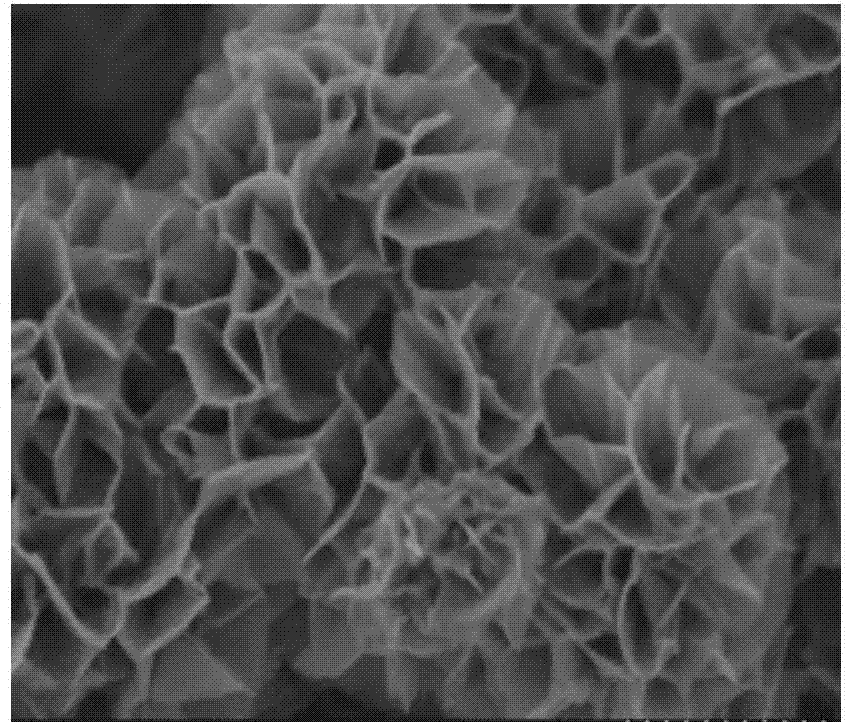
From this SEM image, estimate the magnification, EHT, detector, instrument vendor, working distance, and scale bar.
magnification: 60 K X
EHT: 2 kV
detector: SE(U)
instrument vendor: Hitachi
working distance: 4.5 mm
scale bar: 500 nm